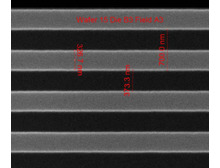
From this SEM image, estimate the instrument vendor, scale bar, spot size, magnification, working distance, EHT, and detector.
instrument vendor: FEI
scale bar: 1000 nm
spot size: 1.5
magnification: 75.019 K X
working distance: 10.2 mm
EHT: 10 kV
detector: ETD